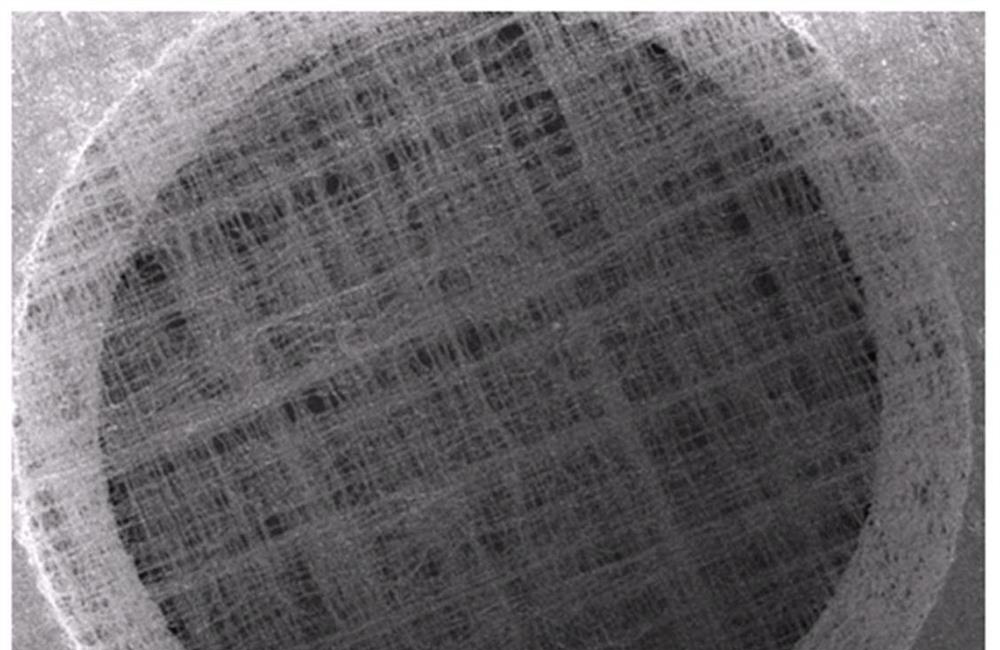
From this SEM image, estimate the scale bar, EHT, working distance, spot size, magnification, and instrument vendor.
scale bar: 20000 nm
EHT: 10 kV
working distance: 6.4 mm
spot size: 3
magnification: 1 K X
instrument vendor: FEI